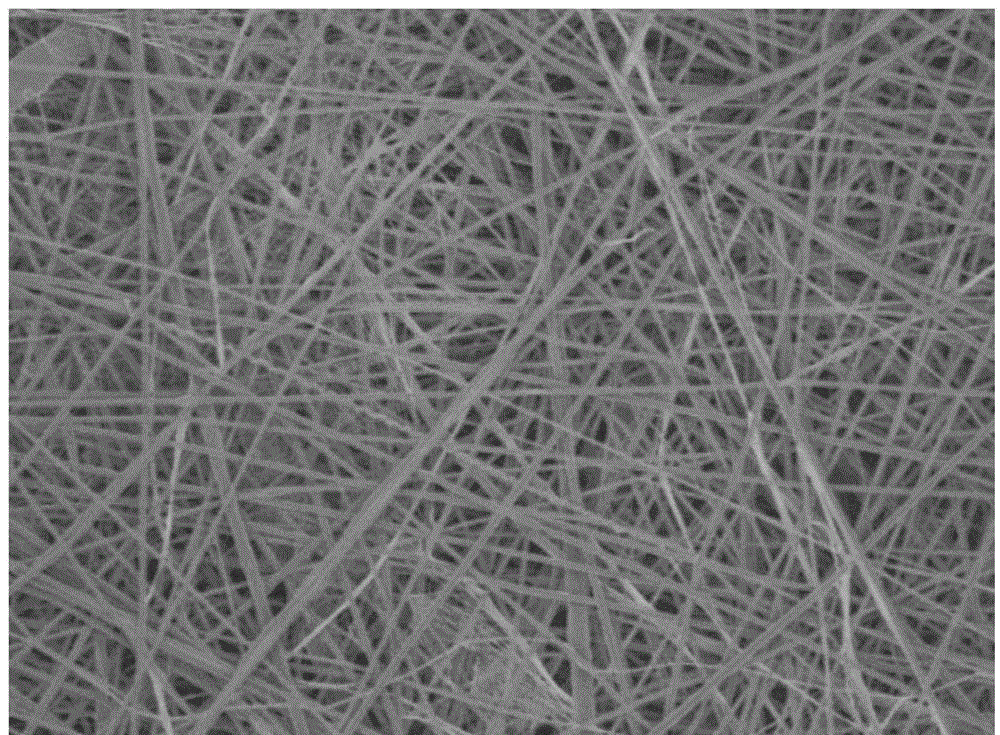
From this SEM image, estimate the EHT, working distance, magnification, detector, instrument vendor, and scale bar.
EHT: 5 kV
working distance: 10.4 mm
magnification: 1 K X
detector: SEI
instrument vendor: JEOL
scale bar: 10000 nm